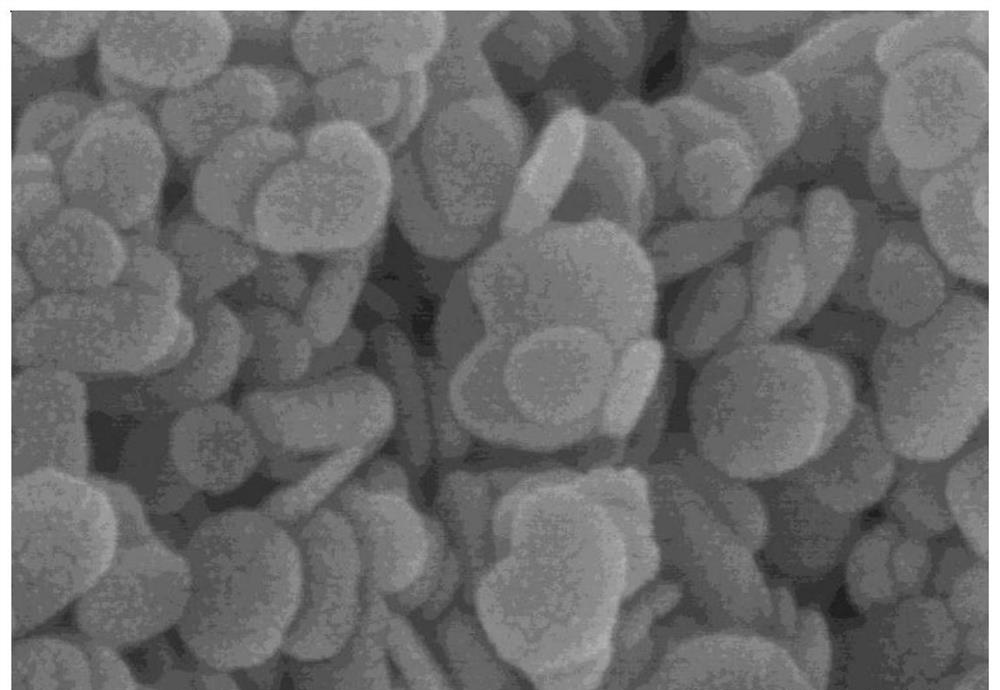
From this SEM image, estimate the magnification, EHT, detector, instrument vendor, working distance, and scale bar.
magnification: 200 K X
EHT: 5 kV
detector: SE(M)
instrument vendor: Hitachi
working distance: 8.8 mm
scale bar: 200 nm